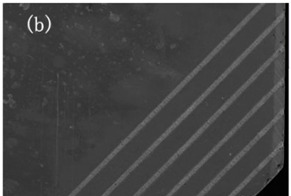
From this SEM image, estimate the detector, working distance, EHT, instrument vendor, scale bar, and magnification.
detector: SE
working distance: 37.1 mm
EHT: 20 kV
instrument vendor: Hitachi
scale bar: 2000000 nm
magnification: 0.02 K X